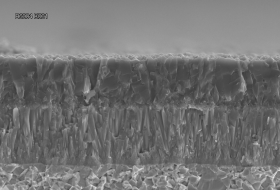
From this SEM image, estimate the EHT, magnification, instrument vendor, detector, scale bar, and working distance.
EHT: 15 kV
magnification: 3 K X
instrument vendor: Hitachi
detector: SE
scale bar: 10000 nm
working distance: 5.6 mm